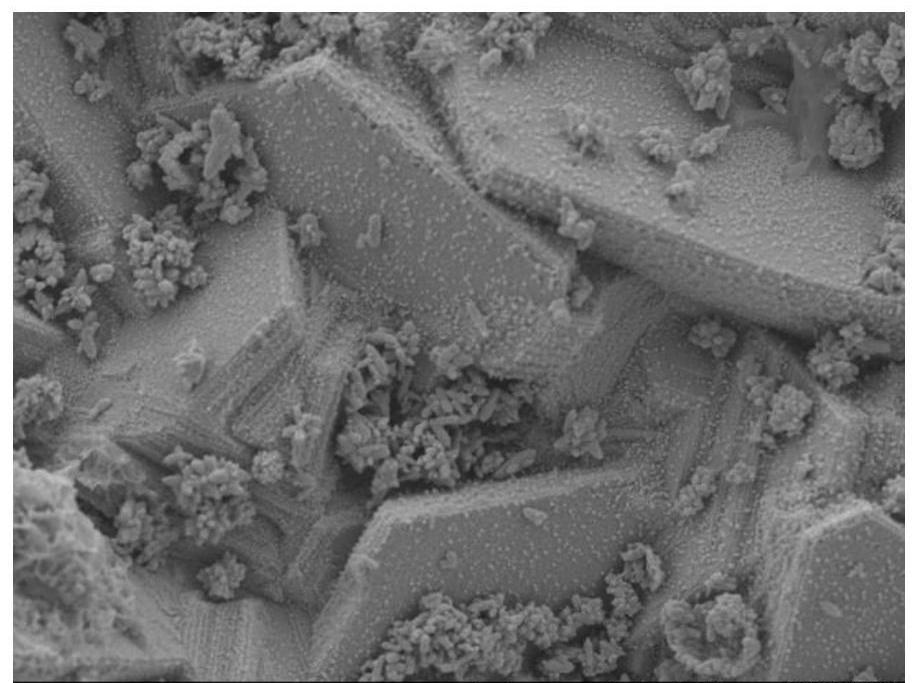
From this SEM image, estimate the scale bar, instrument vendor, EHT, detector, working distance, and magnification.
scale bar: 1000 nm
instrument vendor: JEOL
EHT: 5 kV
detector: LED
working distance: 9.6 mm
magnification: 4 K X